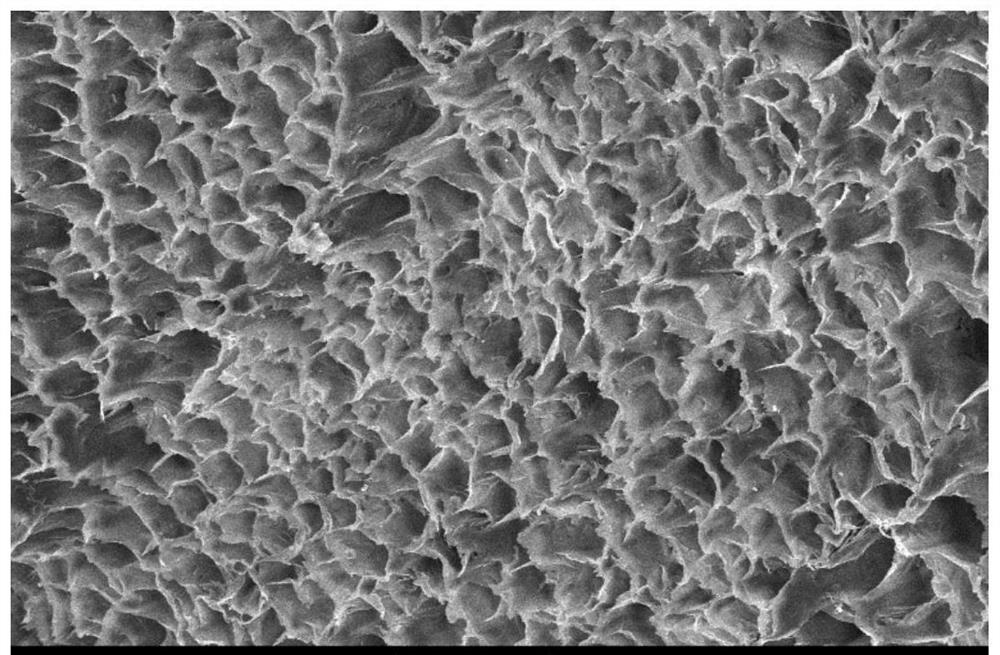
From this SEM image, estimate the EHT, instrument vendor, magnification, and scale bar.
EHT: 15 kV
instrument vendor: JEOL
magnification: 0.2 K X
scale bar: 100000 nm